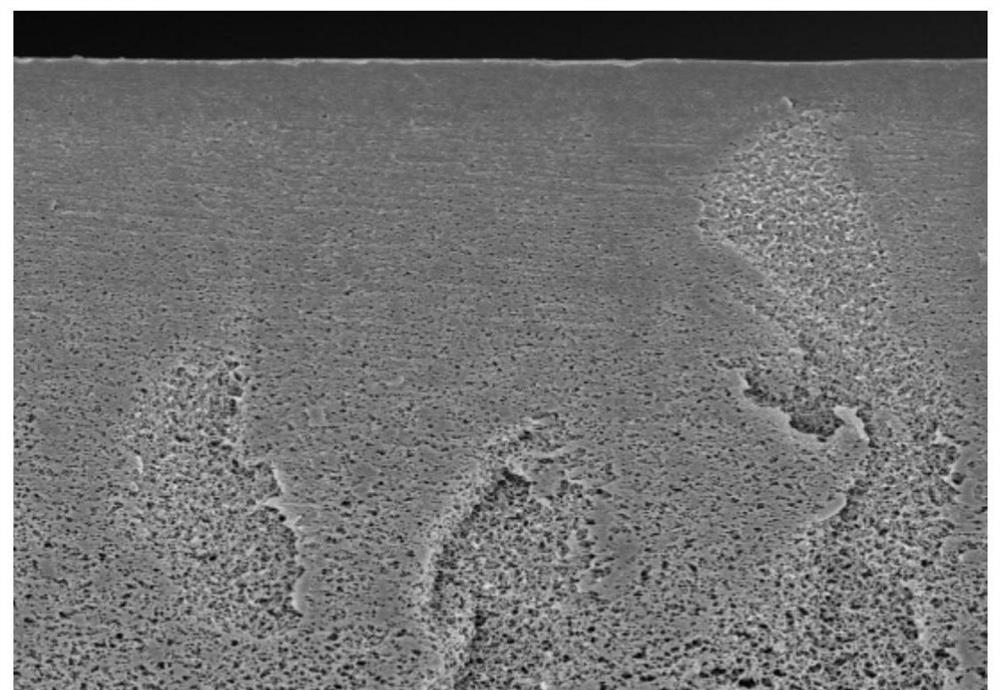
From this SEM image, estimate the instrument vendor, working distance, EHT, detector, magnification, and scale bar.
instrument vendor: Hitachi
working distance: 7.5 mm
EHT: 5 kV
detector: SE(M)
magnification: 3 K X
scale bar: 10000 nm